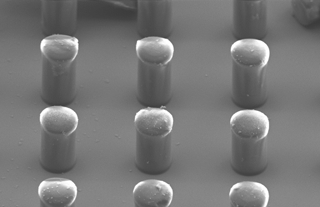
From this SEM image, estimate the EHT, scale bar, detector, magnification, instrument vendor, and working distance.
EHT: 10 kV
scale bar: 20000 nm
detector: SE2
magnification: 0.72 K X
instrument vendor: Zeiss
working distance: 6 mm